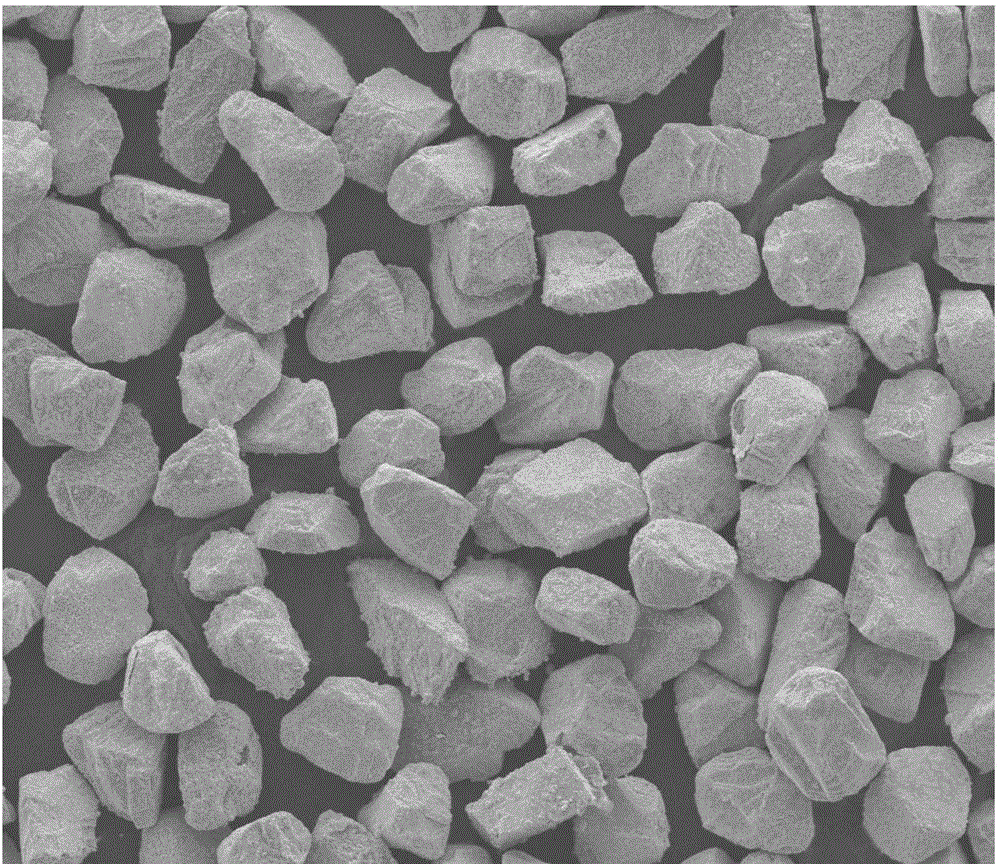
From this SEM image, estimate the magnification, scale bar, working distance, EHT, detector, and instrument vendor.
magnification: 0.5 K X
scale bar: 200000 nm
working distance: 10 mm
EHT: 20 kV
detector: ETD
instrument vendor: FEI